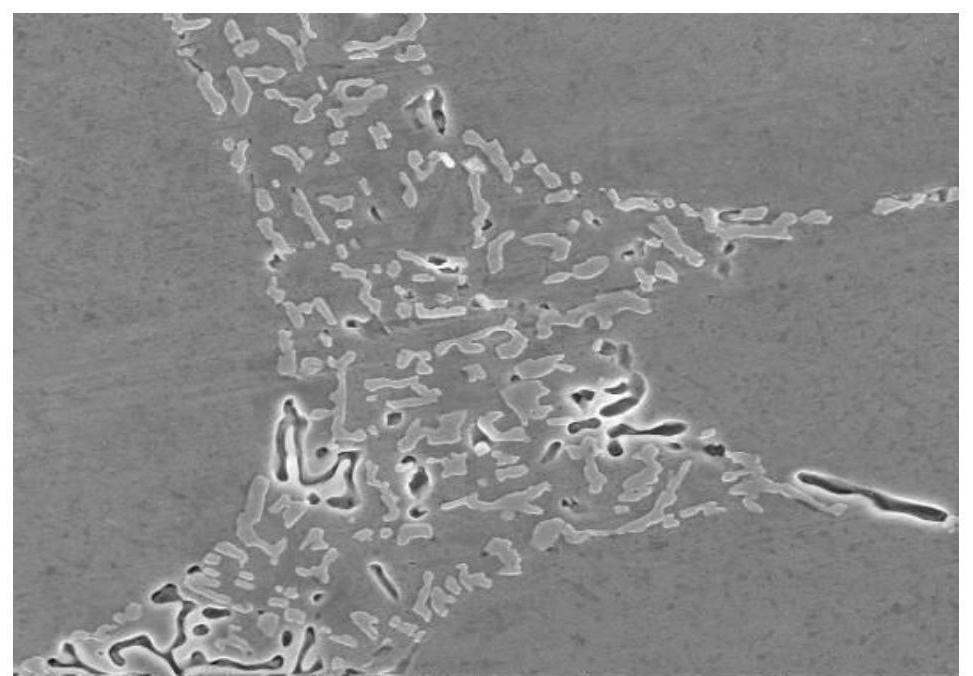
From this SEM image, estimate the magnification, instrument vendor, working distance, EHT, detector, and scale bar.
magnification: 5 K X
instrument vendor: FEI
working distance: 4 mm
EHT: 10 kV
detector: ETD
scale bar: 20000 nm